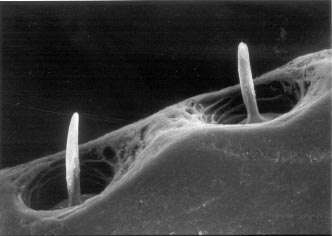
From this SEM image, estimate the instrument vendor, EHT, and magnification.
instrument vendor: JEOL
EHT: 20 kV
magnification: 0.53 K X